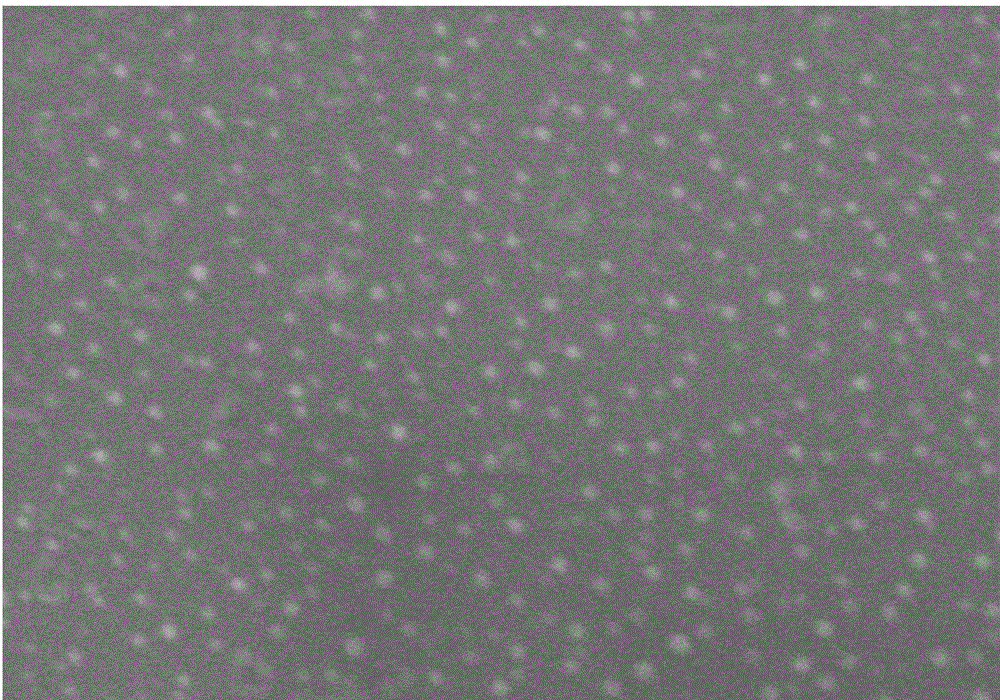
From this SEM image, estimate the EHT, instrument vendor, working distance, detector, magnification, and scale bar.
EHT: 5 kV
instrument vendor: Hitachi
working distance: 15.1 mm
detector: SE(M)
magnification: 70 K X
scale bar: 500 nm